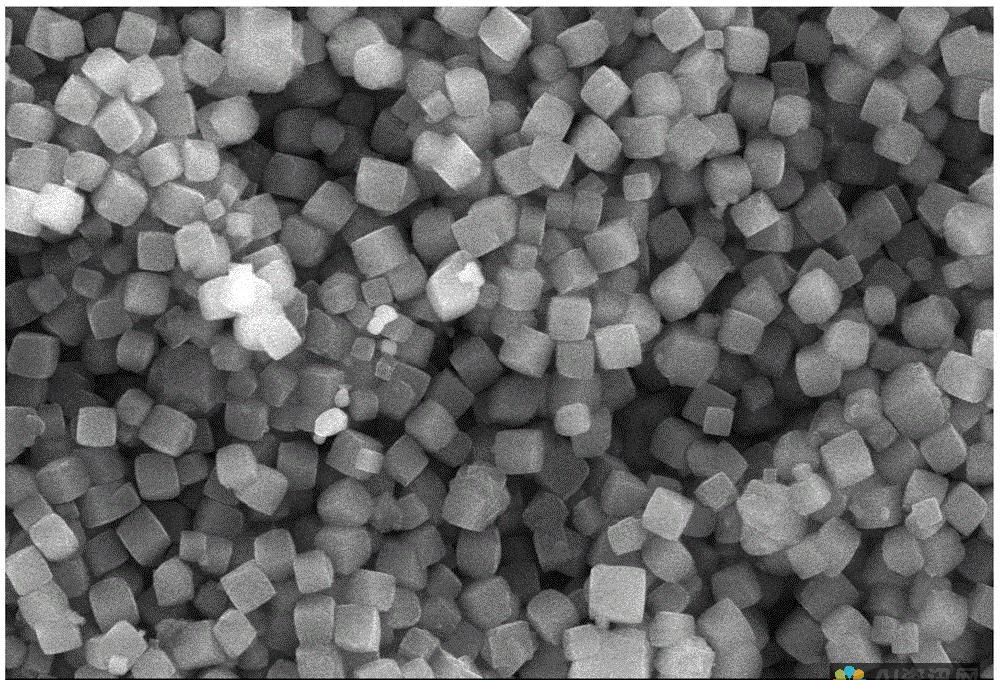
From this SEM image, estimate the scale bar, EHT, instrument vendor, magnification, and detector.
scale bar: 5000 nm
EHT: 20 kV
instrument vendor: JEOL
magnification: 5 K X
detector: SEI